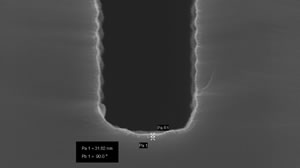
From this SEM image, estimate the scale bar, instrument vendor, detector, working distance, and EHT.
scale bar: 100 nm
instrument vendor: Zeiss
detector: InLens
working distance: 1 mm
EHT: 5 kV